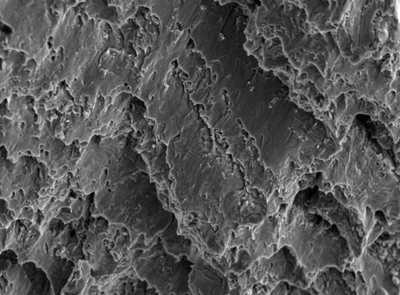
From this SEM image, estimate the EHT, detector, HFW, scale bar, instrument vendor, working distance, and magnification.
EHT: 20 kV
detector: SE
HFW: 139 µm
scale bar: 20000 nm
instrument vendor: Tescan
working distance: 5.07 mm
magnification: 1.99 K X